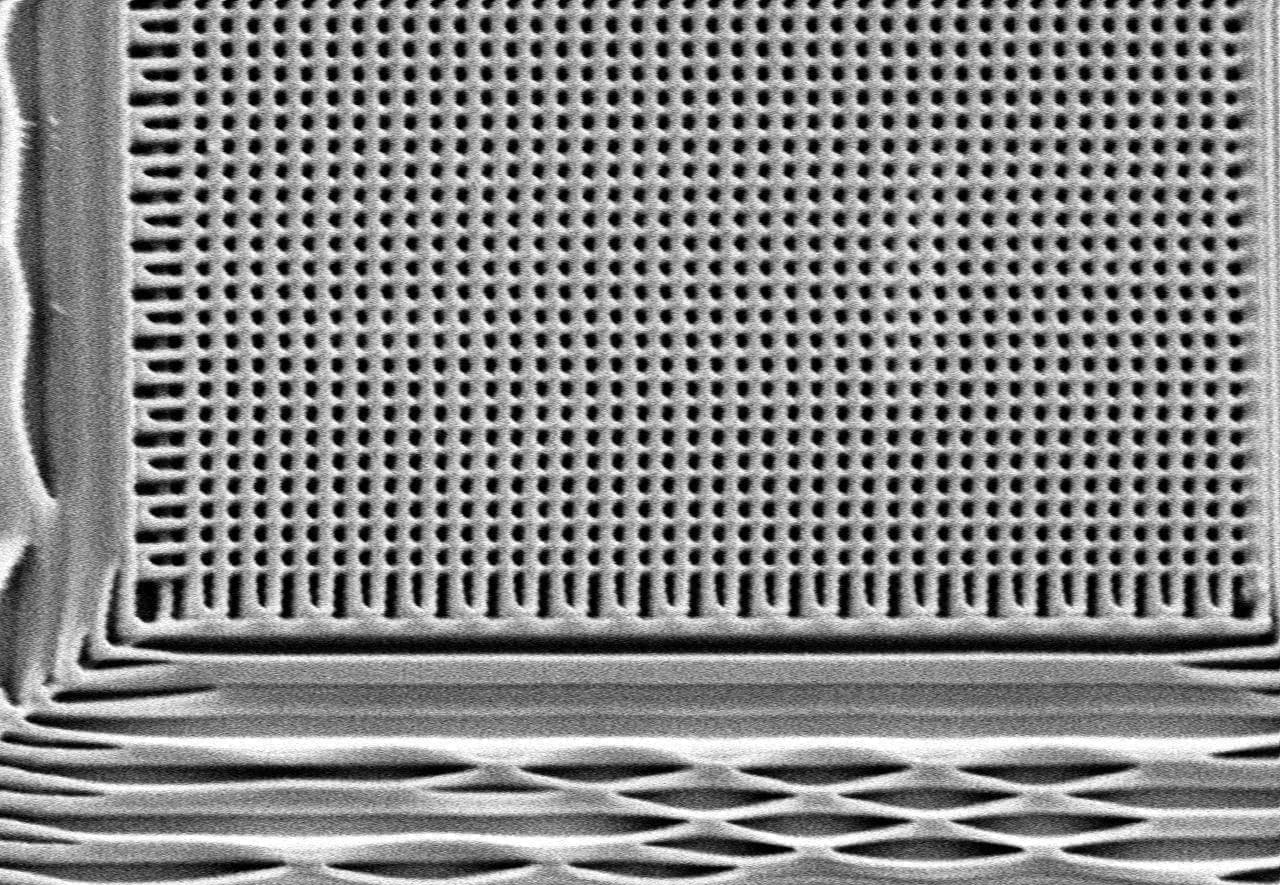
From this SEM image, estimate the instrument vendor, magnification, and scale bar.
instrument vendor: Hitachi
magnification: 2.9 K X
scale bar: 3440 nm